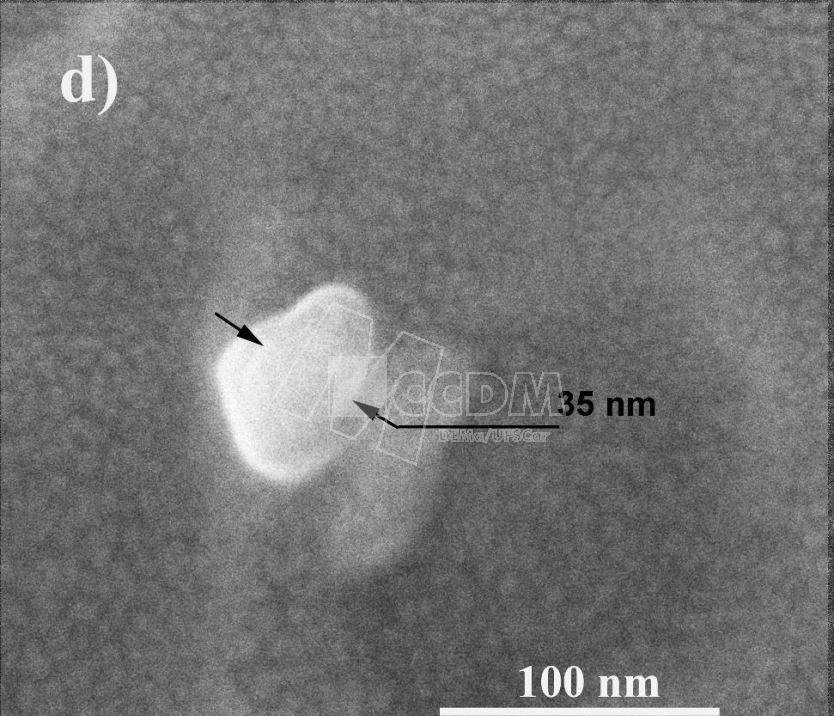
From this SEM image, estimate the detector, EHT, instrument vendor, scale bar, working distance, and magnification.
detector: SE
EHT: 5 kV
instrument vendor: FEI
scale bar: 100 nm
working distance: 5.8 mm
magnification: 500 K X